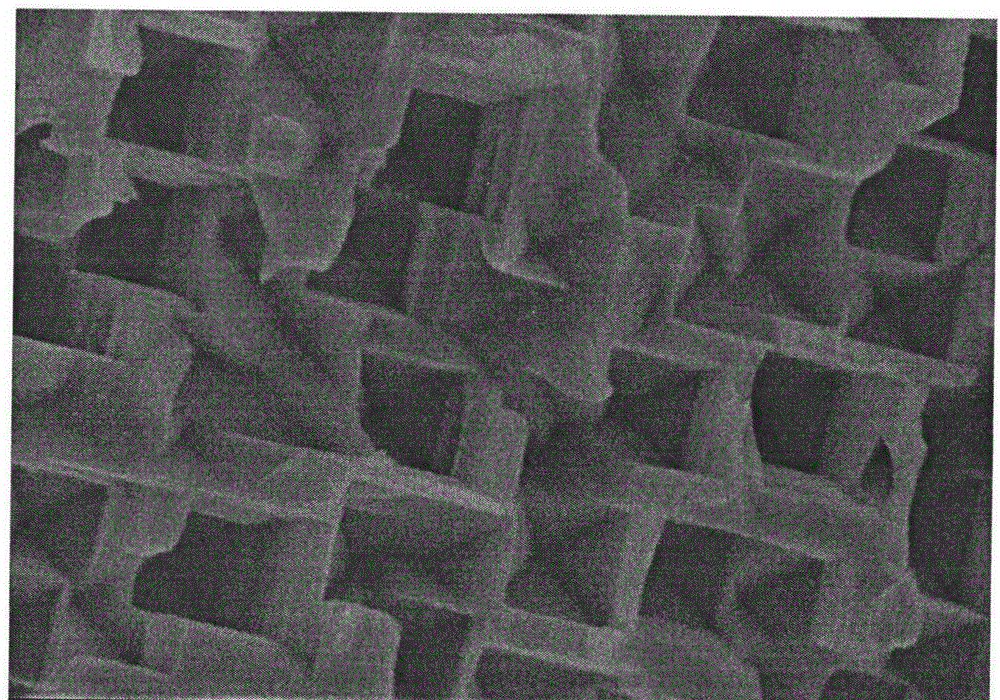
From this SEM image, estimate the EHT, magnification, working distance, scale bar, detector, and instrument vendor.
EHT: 3 kV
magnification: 60 K X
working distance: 7.8 mm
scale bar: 500 nm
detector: SE(M,LA3)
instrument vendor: Hitachi